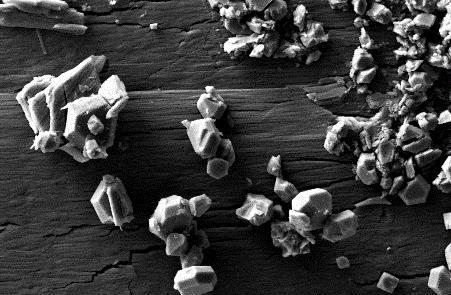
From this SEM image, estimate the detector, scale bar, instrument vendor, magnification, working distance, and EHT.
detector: SE2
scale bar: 2000 nm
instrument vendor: Zeiss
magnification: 19.52 K X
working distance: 3.3 mm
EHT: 5 kV